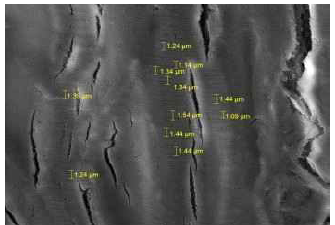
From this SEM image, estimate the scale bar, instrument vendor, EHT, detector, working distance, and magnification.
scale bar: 10000 nm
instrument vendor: Tescan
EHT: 10 kV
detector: SE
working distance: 10.93 mm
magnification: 5 K X